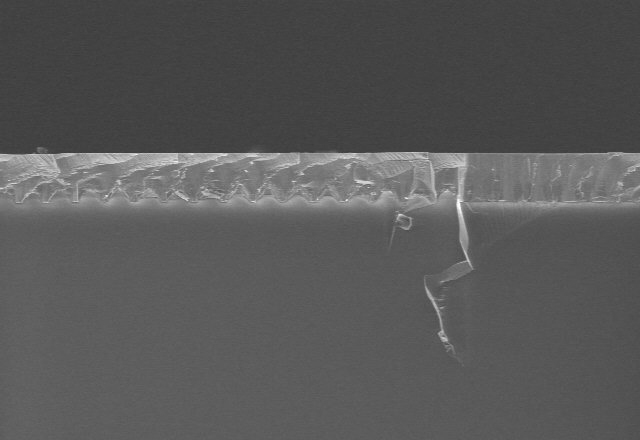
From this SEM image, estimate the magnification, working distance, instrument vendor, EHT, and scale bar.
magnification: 2 K X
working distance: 10.3 mm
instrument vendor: Hitachi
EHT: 5 kV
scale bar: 20000 nm